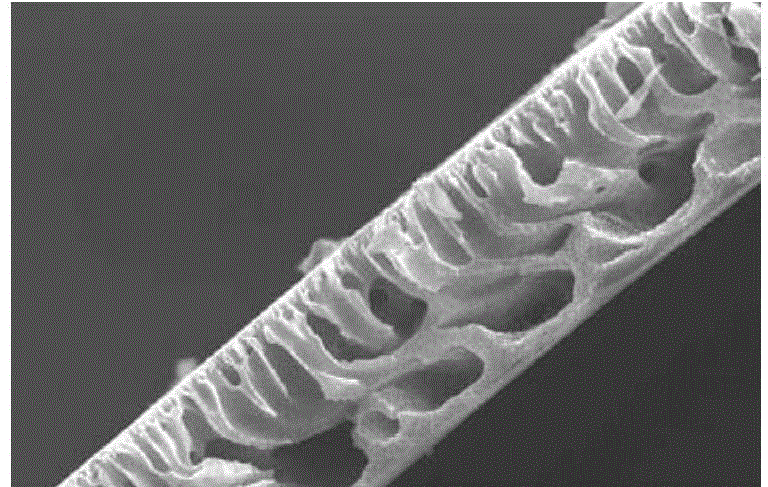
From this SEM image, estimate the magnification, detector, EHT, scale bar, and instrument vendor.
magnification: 0.3 K X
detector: SEI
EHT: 30 kV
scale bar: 50000 nm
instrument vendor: JEOL